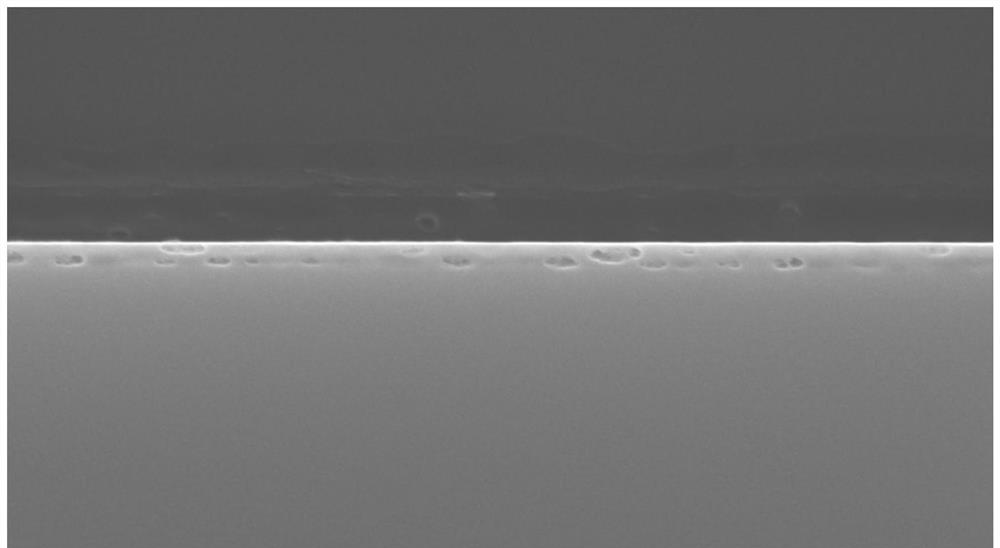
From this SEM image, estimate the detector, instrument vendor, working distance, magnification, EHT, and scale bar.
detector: SE(UL)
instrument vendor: Hitachi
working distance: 8.6 mm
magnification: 50 K X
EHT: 15 kV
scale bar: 1000 nm